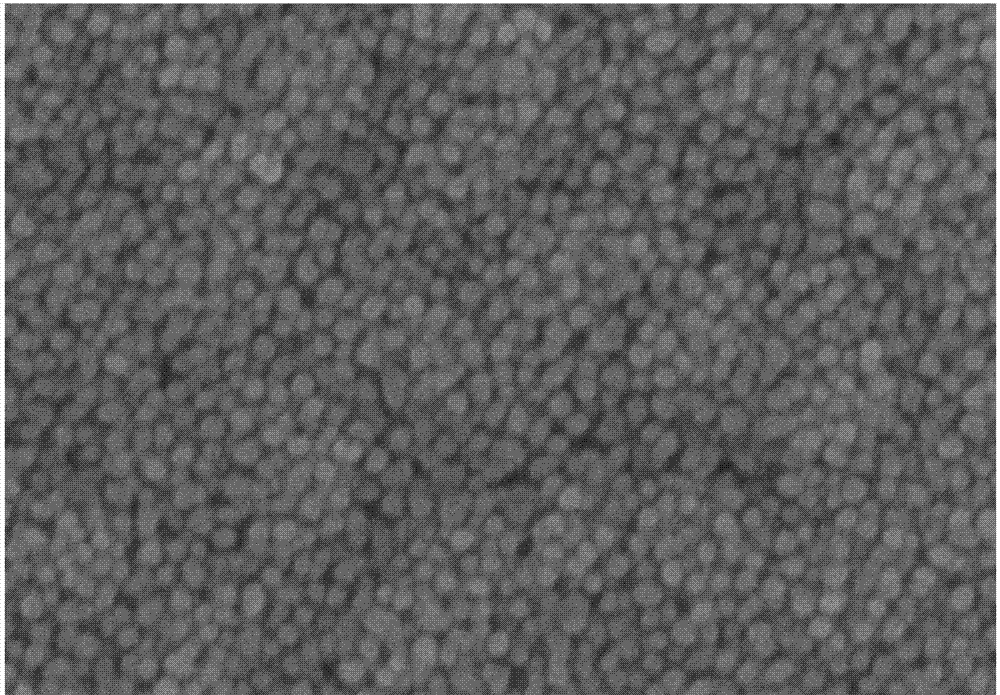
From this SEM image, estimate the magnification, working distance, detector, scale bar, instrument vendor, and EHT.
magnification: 200 K X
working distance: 6.7 mm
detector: SE(M)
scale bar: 200 nm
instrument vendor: Hitachi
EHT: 5 kV